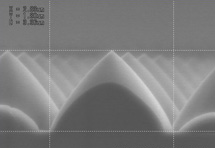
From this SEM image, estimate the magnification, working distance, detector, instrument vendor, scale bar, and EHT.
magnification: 25 K X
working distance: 9.2 mm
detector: SE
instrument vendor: Hitachi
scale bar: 2000 nm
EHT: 5 kV